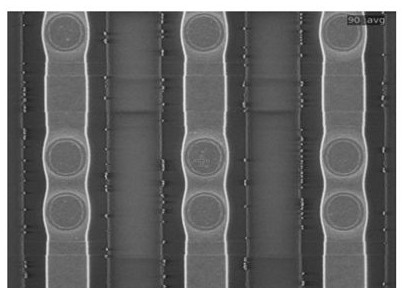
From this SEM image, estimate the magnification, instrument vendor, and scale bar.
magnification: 2.4 K X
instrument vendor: other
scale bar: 10000 nm